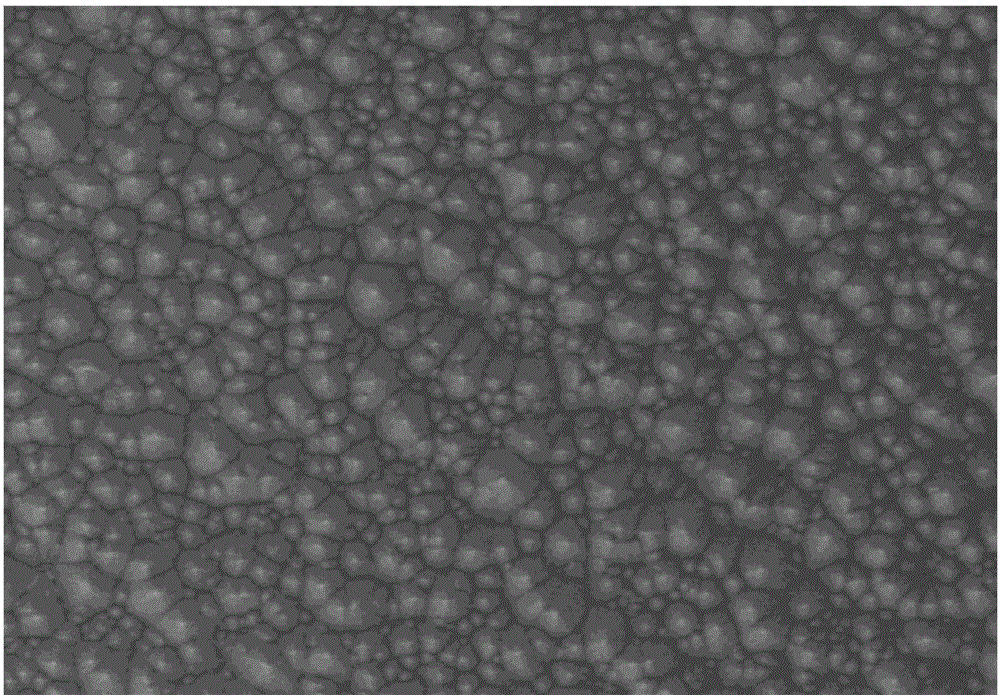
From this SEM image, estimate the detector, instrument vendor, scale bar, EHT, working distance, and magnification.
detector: SE(UL)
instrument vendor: Hitachi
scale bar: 50000 nm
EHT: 10 kV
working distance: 8.5 mm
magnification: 1 K X